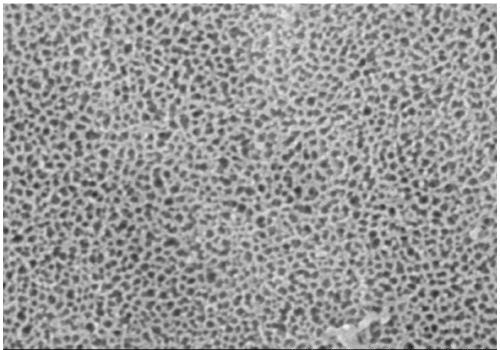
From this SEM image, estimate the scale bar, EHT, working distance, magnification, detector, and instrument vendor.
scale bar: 500 nm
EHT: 5 kV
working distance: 4 mm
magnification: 100 K X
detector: SE(M)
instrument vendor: Hitachi